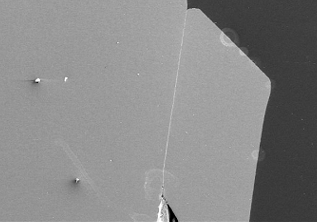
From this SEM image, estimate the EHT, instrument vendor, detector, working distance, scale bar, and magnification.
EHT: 10 kV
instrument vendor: Hitachi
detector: SE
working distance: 13.9 mm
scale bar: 1000000 nm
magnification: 0.05 K X